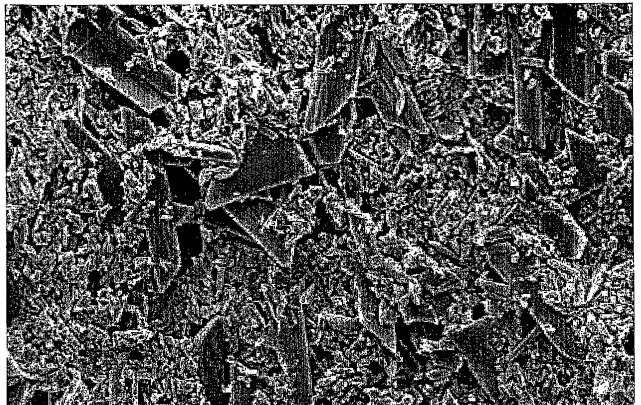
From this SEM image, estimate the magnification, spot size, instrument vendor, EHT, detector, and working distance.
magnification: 10 K X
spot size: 2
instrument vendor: FEI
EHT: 3 kV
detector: SE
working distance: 9.9 mm